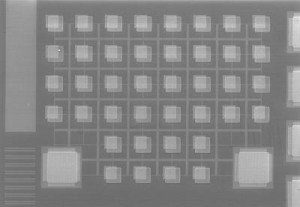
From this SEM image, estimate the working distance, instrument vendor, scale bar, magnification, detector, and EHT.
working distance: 12 mm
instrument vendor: Hitachi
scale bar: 300000 nm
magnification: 0.18 K X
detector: SE(M)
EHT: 20 kV